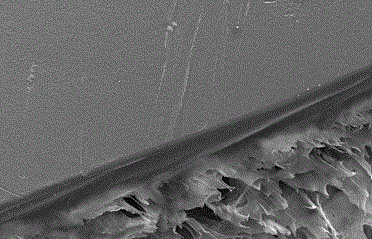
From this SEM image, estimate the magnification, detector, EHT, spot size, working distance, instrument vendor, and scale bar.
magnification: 0.5 K X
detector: SE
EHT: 25 kV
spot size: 4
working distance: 6.6 mm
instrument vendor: FEI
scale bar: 100000 nm